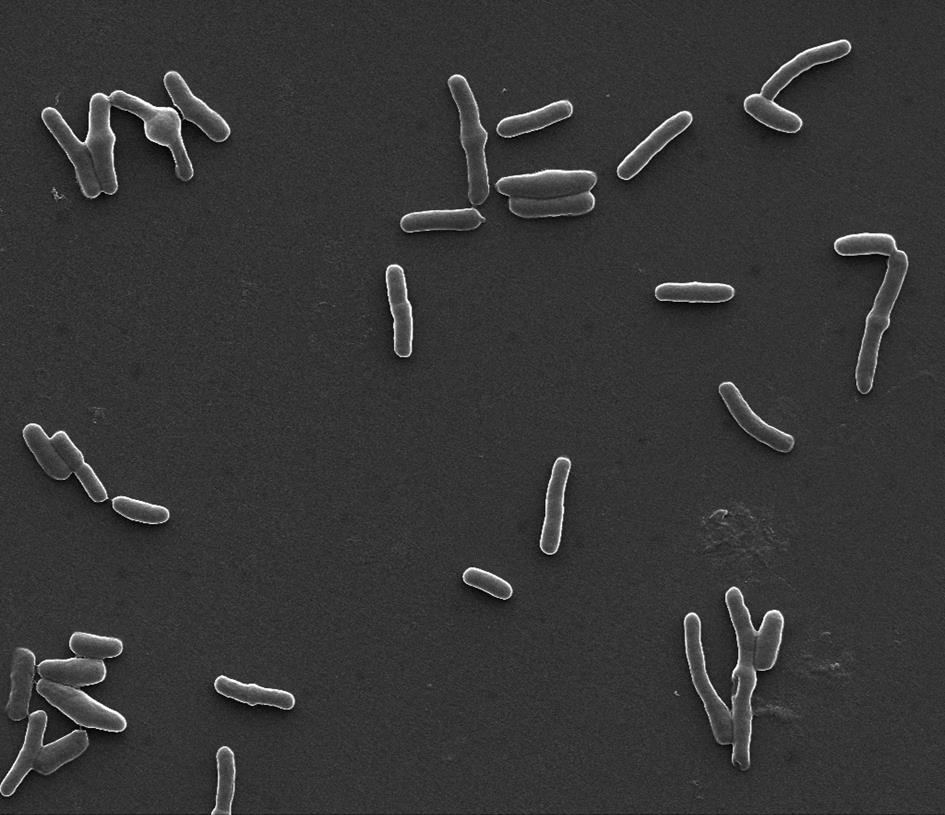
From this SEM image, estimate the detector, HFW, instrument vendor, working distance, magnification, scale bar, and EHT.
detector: SE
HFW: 32 µm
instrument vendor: FEI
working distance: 9.7 mm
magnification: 8 K X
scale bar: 10000 nm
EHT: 30 kV